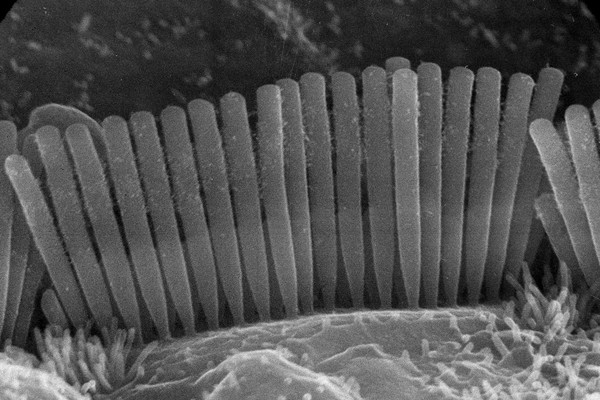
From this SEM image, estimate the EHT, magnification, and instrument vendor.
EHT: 25 kV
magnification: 11 K X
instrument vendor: JEOL